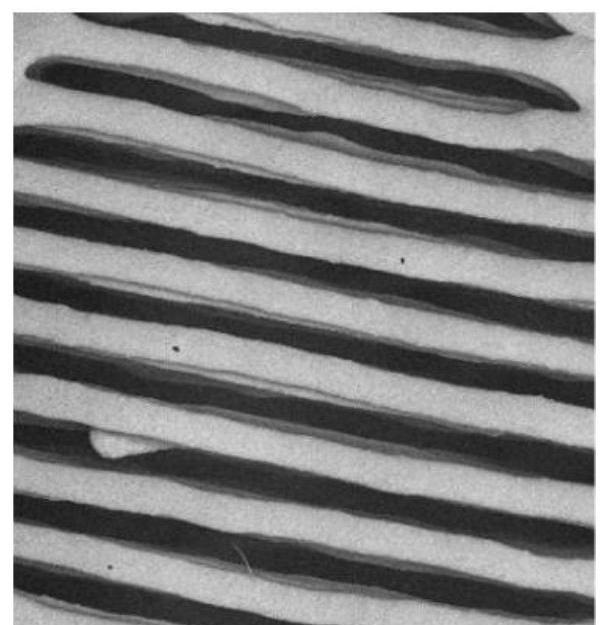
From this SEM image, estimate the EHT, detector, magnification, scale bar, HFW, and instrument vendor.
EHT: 8 kV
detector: BSD Full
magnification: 0.057 K X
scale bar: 2000000 nm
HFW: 5640 µm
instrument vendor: Thermo